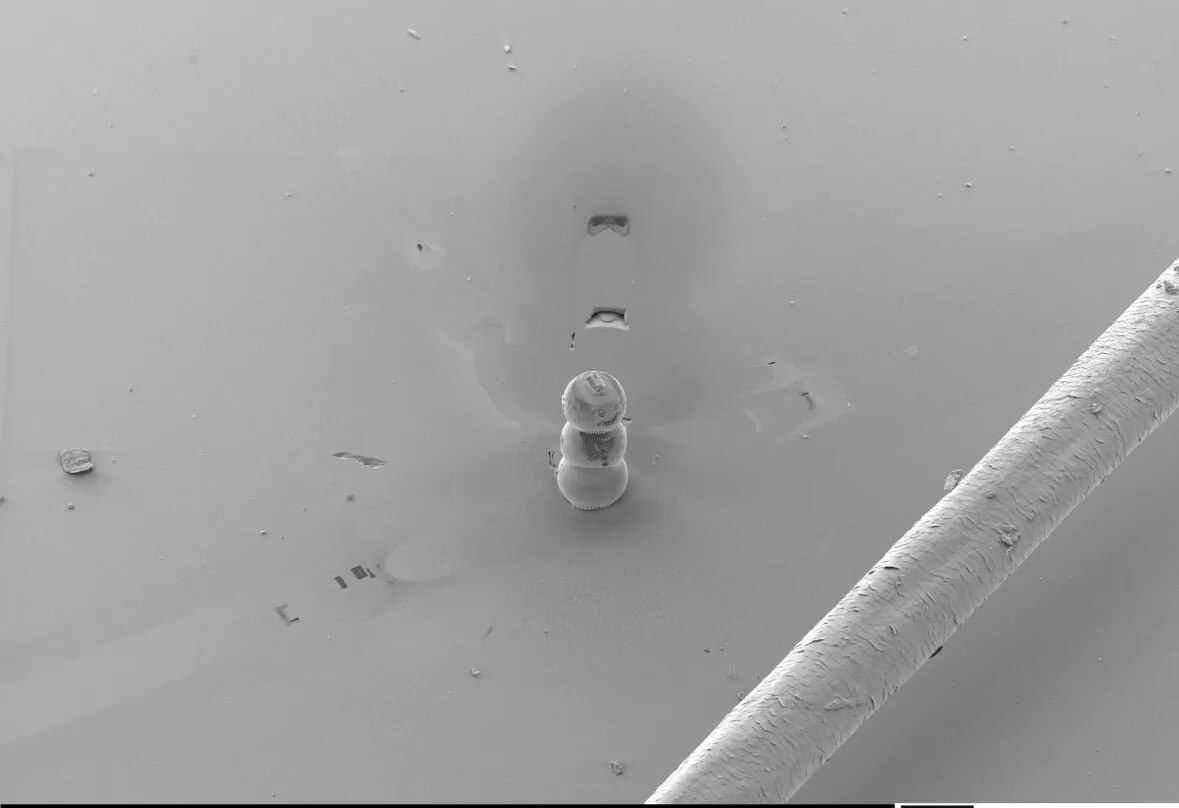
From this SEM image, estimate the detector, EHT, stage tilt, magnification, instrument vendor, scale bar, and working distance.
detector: SESI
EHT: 7 kV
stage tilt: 40°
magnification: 0.125 K X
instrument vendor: Zeiss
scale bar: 20000 nm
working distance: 6.8 mm